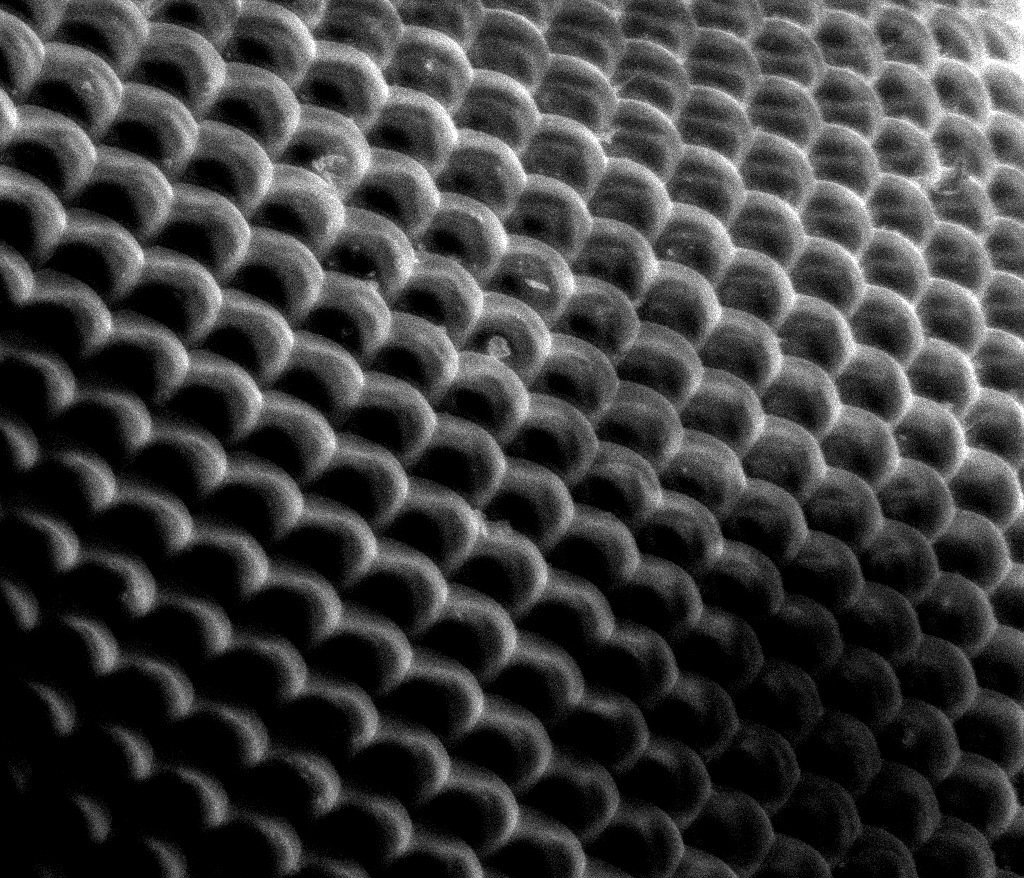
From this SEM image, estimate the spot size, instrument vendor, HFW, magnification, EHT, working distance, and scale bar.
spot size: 2.5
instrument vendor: FEI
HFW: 260 µm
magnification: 1.043 K X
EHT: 10 kV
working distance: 9.3 mm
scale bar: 100000 nm